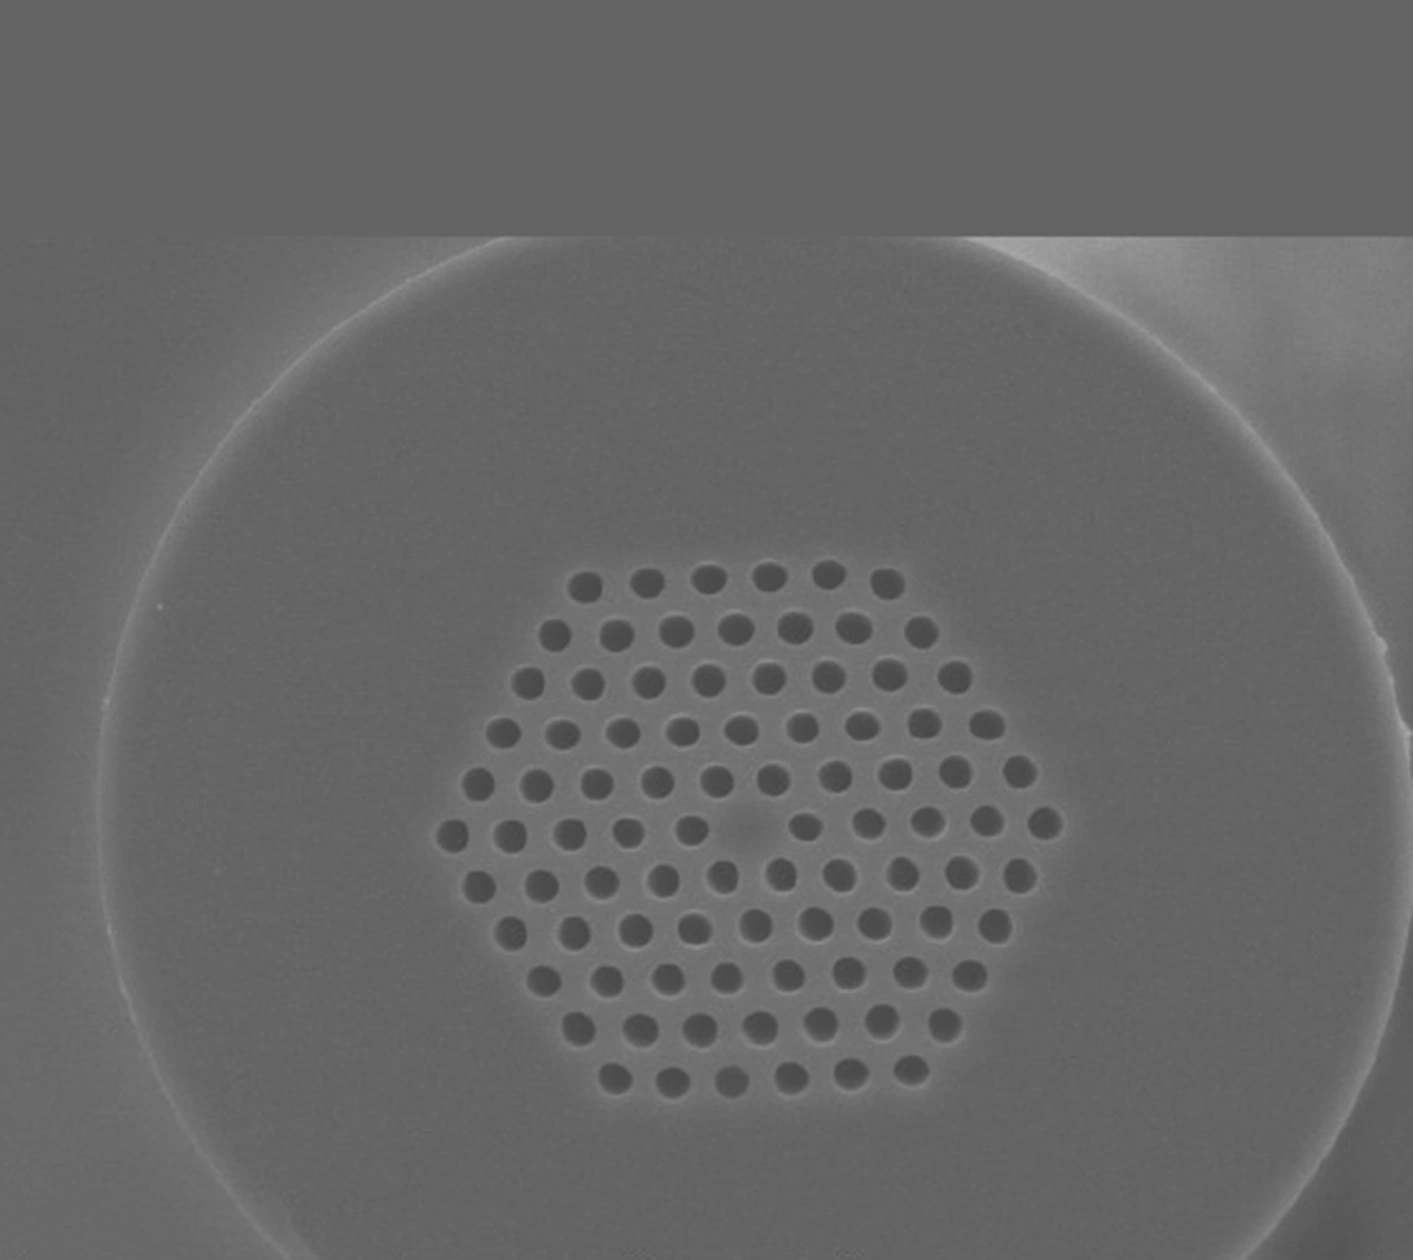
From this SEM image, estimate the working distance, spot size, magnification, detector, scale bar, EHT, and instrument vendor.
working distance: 14.6 mm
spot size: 4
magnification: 0.8 K X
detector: SE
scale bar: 20000 nm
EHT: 20 kV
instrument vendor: FEI